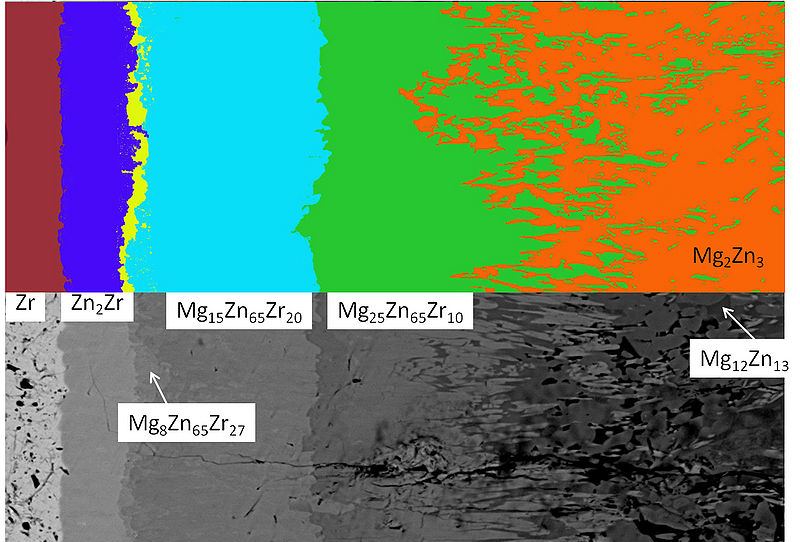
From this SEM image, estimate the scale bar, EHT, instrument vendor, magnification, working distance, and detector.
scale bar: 50000 nm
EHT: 15 kV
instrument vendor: Hitachi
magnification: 1 K X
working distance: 10.1 mm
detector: BSECOMP